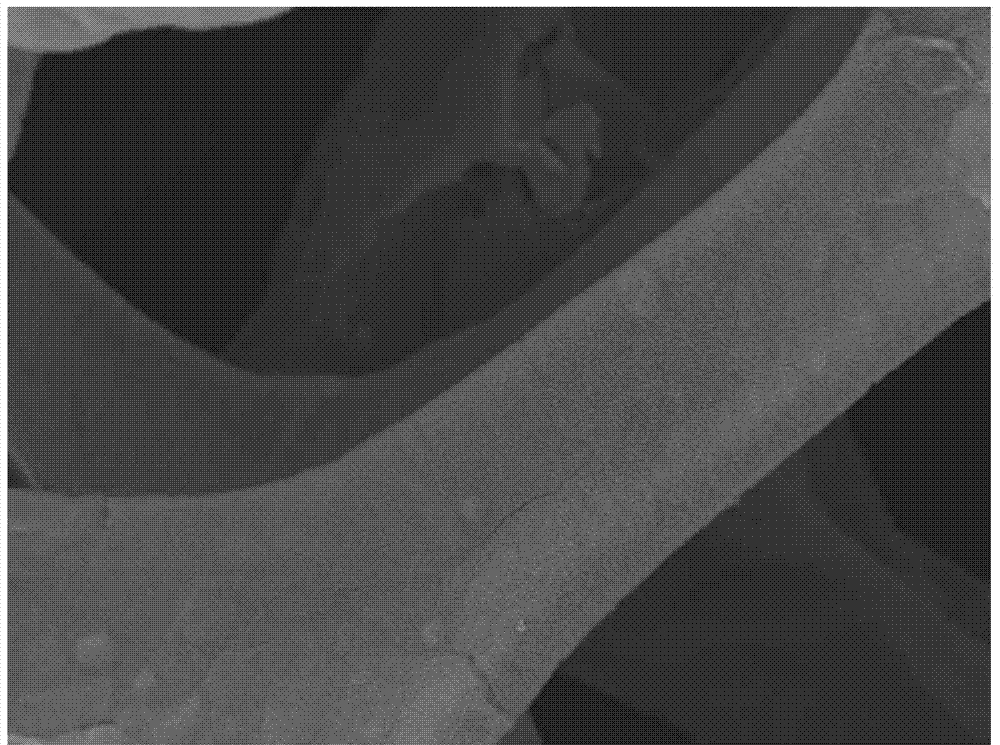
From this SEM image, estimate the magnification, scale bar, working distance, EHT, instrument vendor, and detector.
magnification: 0.5 K X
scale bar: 10000 nm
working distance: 10.3 mm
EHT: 15 kV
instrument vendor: JEOL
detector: SEI